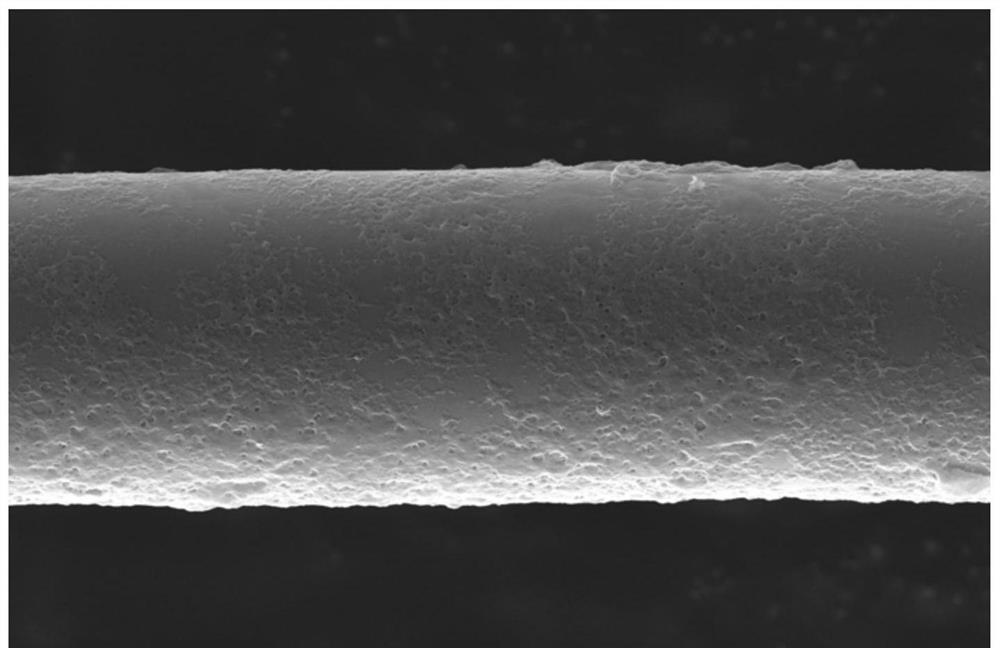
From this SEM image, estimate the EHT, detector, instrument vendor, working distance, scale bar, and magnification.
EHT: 15 kV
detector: ETD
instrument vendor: FEI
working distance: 8.1 mm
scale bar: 5000 nm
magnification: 15 K X